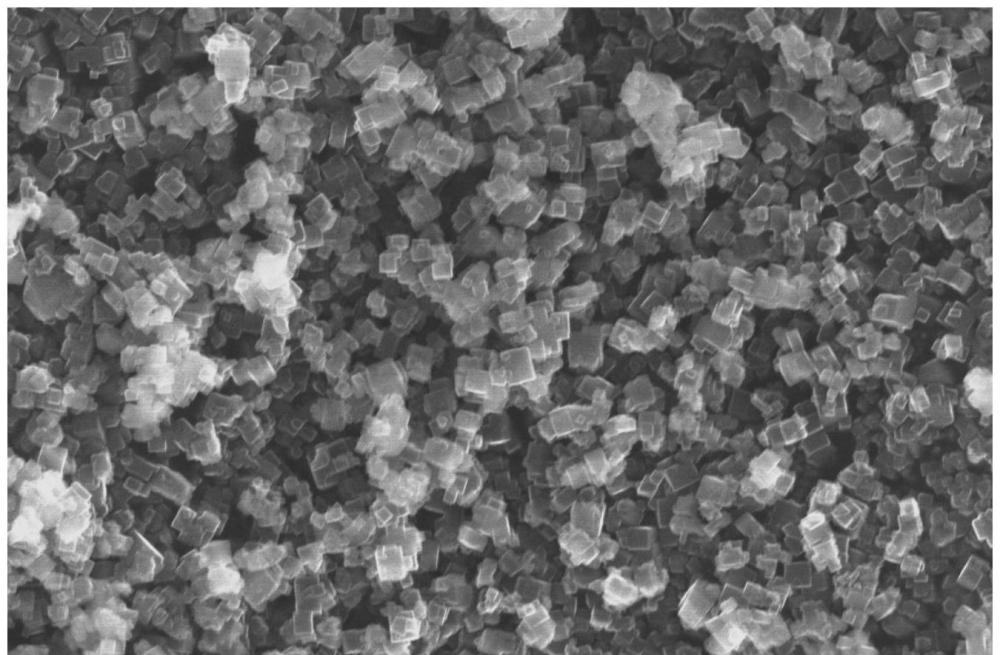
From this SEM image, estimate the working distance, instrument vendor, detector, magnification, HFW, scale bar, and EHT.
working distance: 14.2 mm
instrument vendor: Thermo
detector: ETD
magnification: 30 K X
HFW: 13.8 µm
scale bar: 5000 nm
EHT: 30 kV